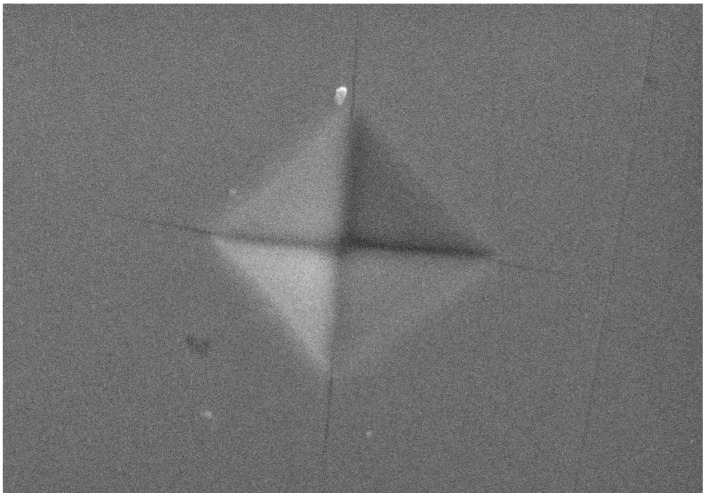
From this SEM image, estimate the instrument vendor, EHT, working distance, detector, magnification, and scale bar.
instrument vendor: Hitachi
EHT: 20 kV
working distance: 20.7 mm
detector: SE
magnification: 3 K X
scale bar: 10000 nm